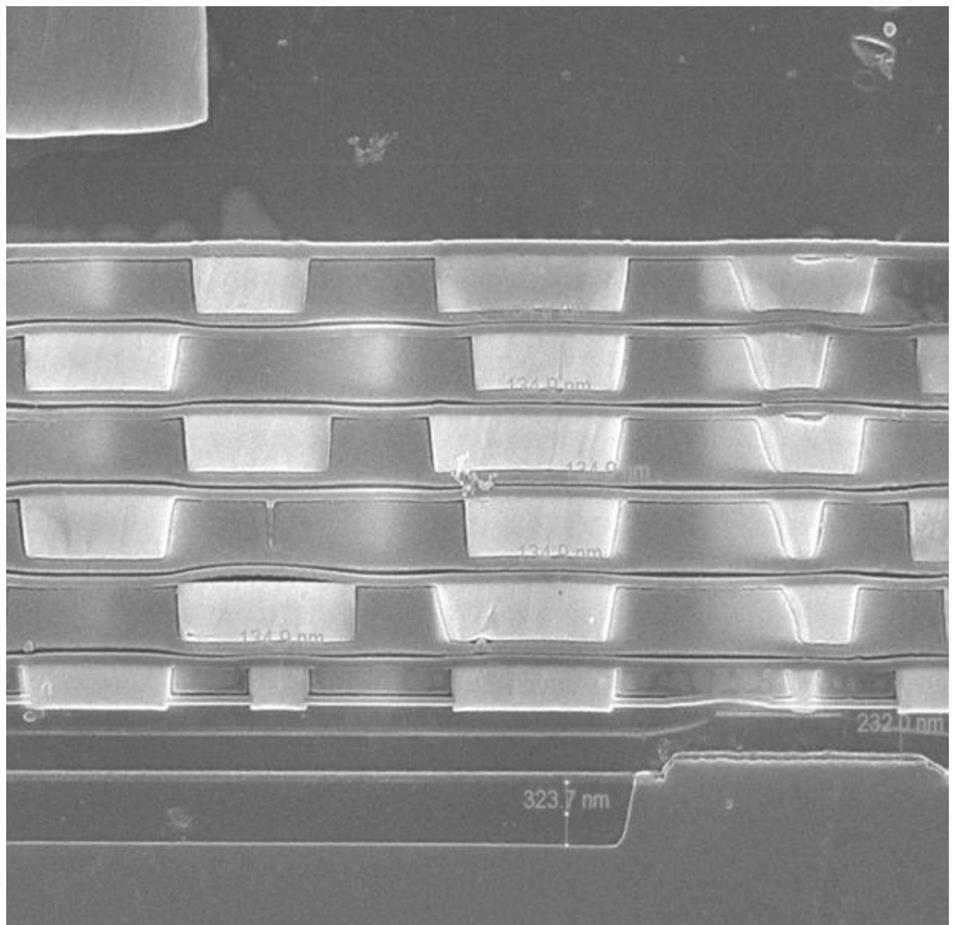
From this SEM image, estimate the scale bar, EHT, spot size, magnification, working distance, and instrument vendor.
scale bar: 2000 nm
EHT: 10 kV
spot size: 3.5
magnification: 50 K X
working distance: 5.1 mm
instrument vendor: FEI